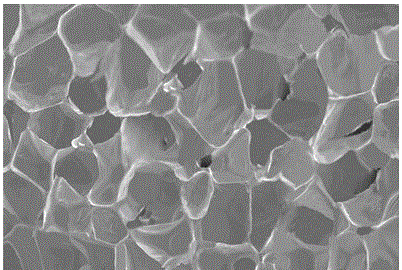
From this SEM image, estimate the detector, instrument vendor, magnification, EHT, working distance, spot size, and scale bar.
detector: ETD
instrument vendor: FEI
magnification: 0.1 K X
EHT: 5 kV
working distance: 12.2 mm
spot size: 3.9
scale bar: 1000000 nm